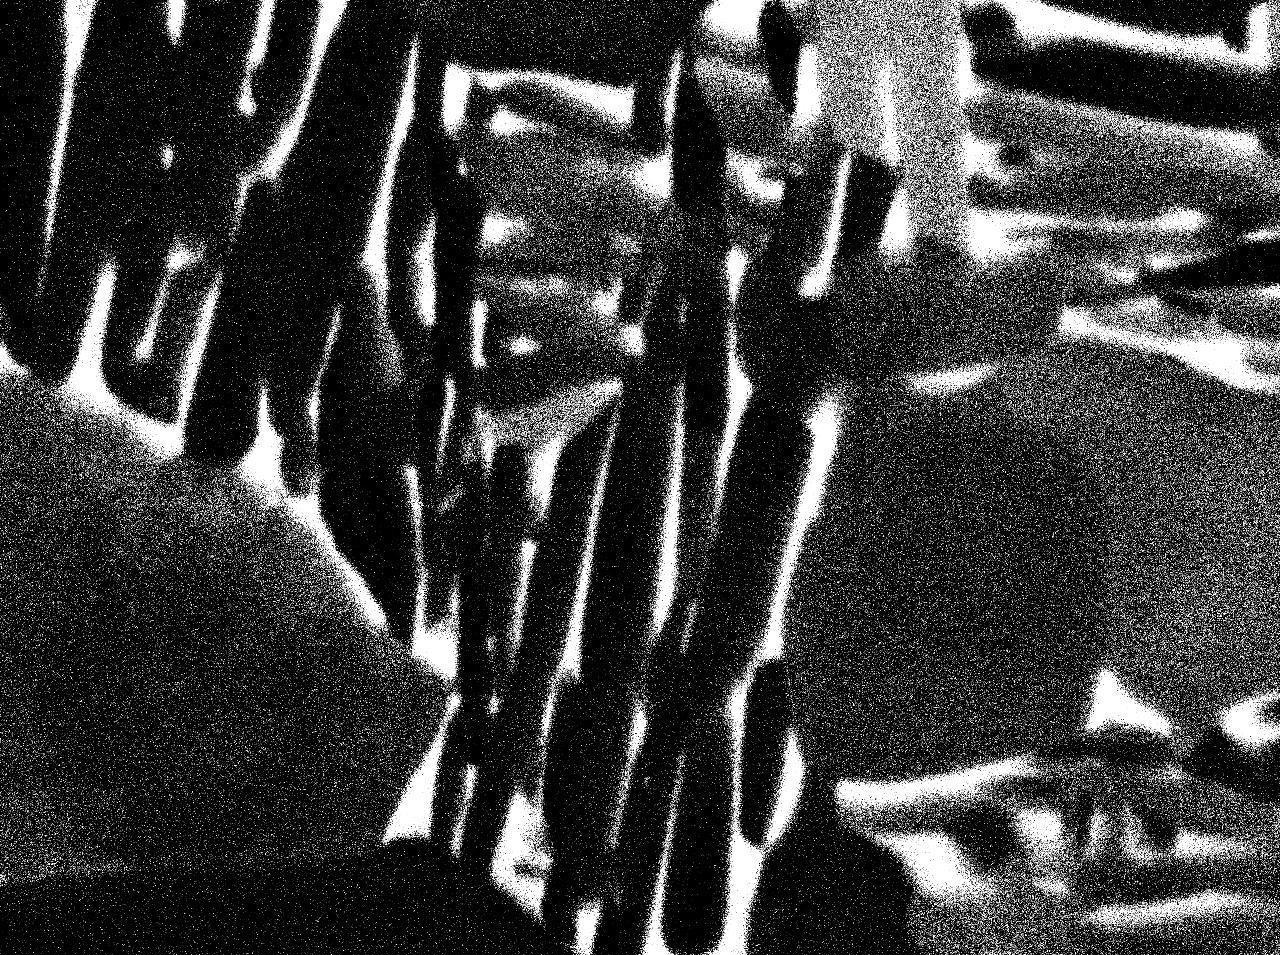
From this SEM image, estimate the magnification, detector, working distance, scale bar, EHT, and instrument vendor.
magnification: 10 K X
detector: COMPO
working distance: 10 mm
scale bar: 1000 nm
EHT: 15 kV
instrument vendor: JEOL